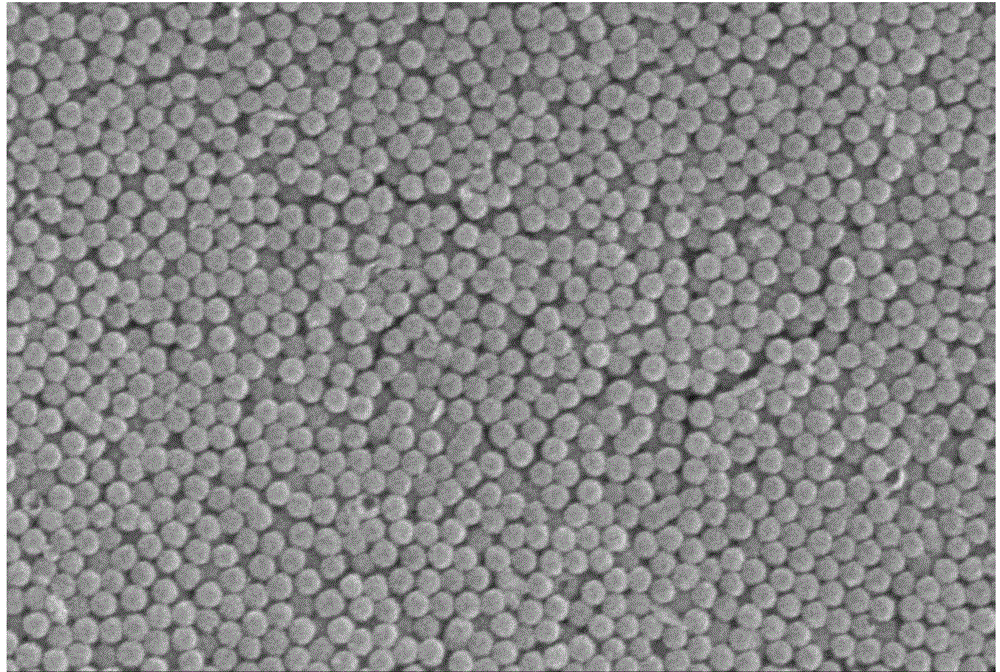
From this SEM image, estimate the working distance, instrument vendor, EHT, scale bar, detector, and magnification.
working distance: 15.8 mm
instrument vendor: Hitachi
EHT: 10 kV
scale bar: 2000 nm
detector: SE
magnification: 20 K X